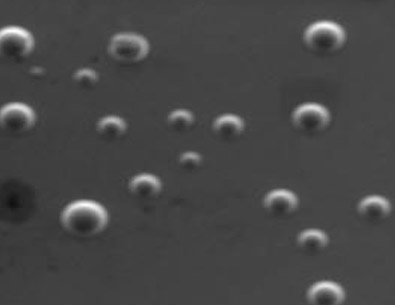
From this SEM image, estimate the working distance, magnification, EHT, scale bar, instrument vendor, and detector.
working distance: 15.57 mm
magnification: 3.08 K X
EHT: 15 kV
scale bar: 20000 nm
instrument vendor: Tescan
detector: SE